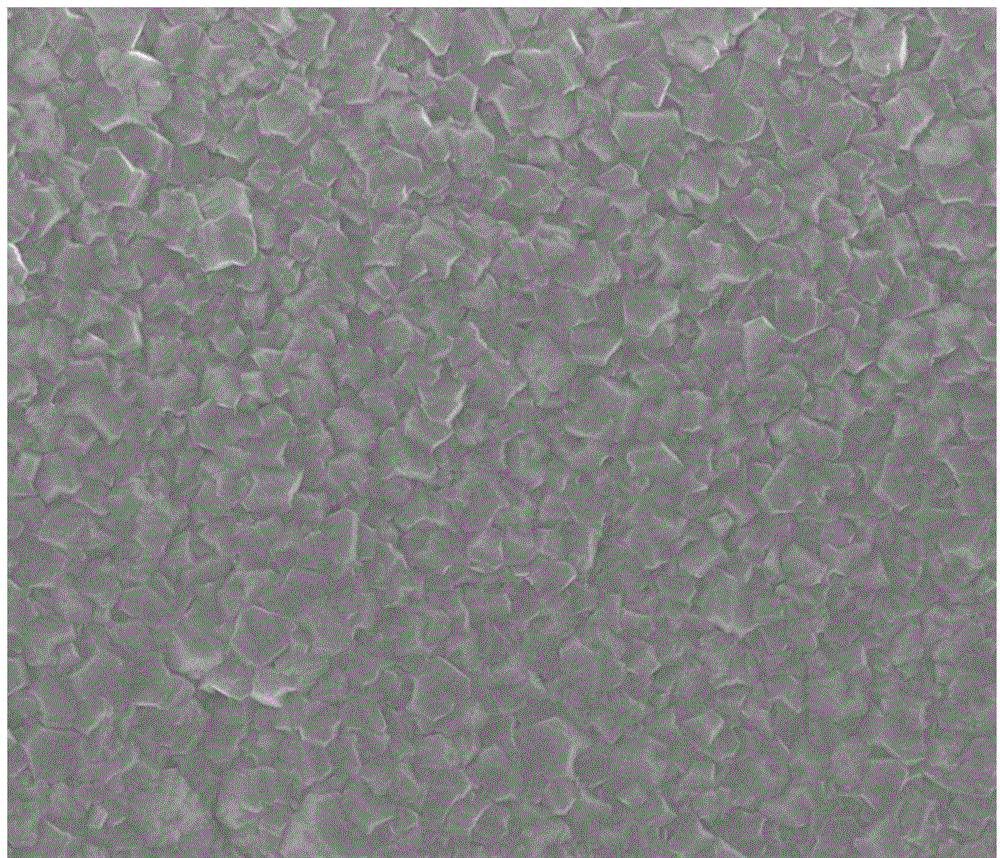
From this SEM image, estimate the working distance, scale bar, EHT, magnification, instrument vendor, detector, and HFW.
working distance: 4.3 mm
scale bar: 5000 nm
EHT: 10 kV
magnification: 10 K X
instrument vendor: FEI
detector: TLD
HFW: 24 µm